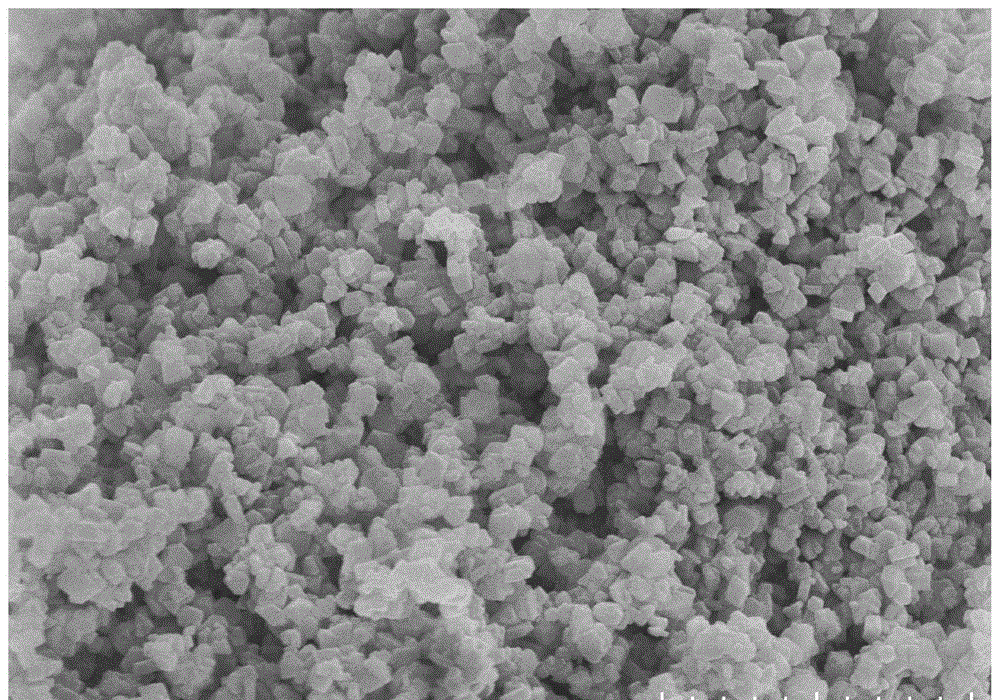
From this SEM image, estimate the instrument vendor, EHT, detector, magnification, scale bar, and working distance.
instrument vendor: Hitachi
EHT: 5 kV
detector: SE(UL)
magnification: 20 K X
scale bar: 2000 nm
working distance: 8.8 mm